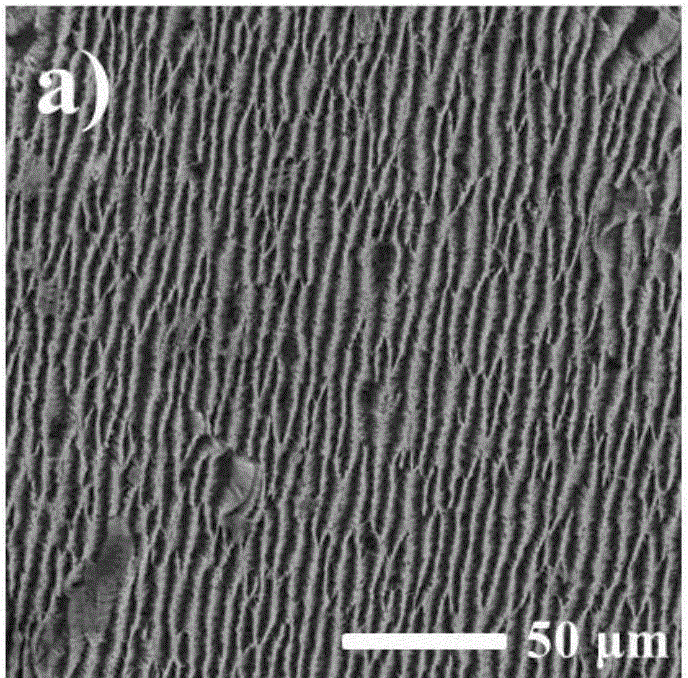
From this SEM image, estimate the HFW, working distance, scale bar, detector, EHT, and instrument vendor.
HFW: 208 µm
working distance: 18.19 mm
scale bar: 50000 nm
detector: SE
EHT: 20 kV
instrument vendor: Tescan